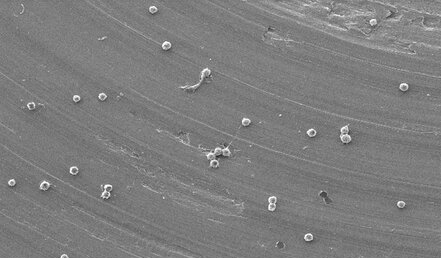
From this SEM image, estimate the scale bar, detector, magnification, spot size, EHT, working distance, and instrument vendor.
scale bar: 50000 nm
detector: SE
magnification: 0.5 K X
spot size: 3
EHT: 10 kV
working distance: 7.3 mm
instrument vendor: FEI